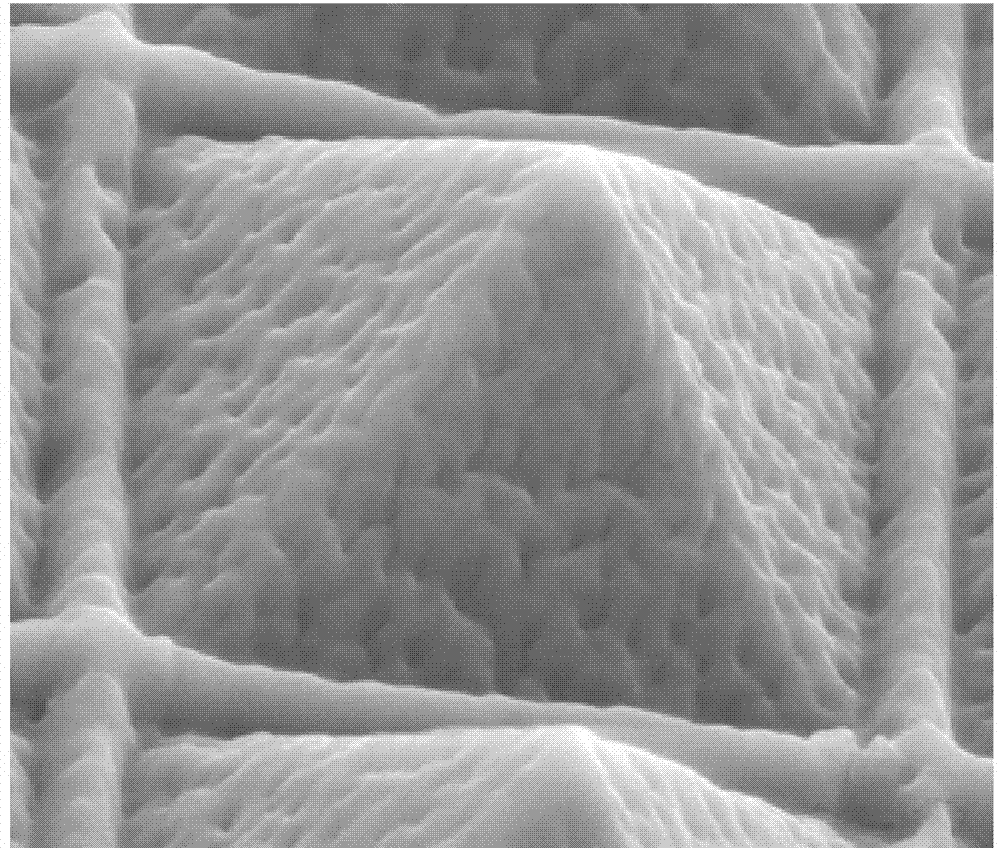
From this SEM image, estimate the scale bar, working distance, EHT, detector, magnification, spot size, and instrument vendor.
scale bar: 5000 nm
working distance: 15.6 mm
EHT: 10 kV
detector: LFD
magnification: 15 K X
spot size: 3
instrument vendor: FEI